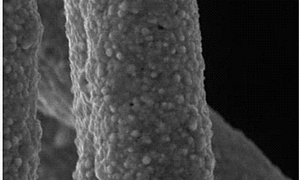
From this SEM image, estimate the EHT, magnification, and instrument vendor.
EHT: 20 kV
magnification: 10 K X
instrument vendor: Tescan